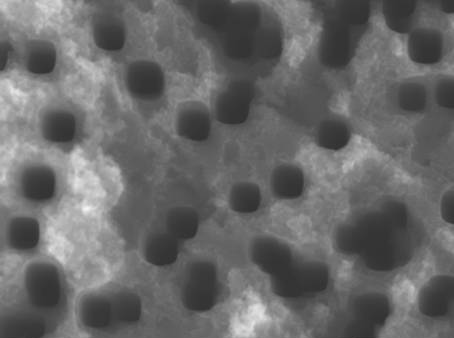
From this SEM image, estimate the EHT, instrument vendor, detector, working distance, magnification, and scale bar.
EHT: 15 kV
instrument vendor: JEOL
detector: SEI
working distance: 6.7 mm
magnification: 30 K X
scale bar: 100 nm